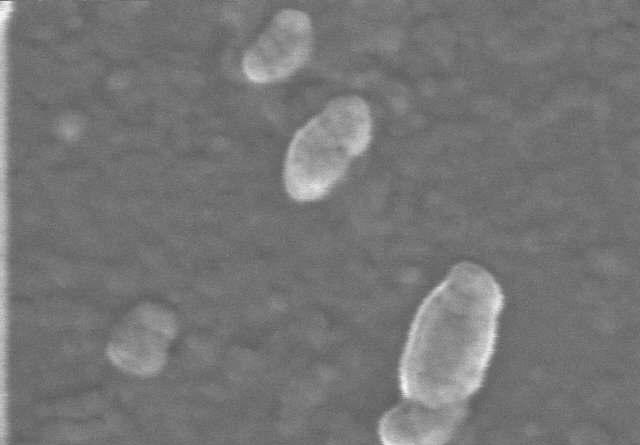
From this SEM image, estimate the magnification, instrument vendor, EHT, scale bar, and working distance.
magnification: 200 K X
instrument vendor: Hitachi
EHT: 15 kV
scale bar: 200 nm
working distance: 12.2 mm